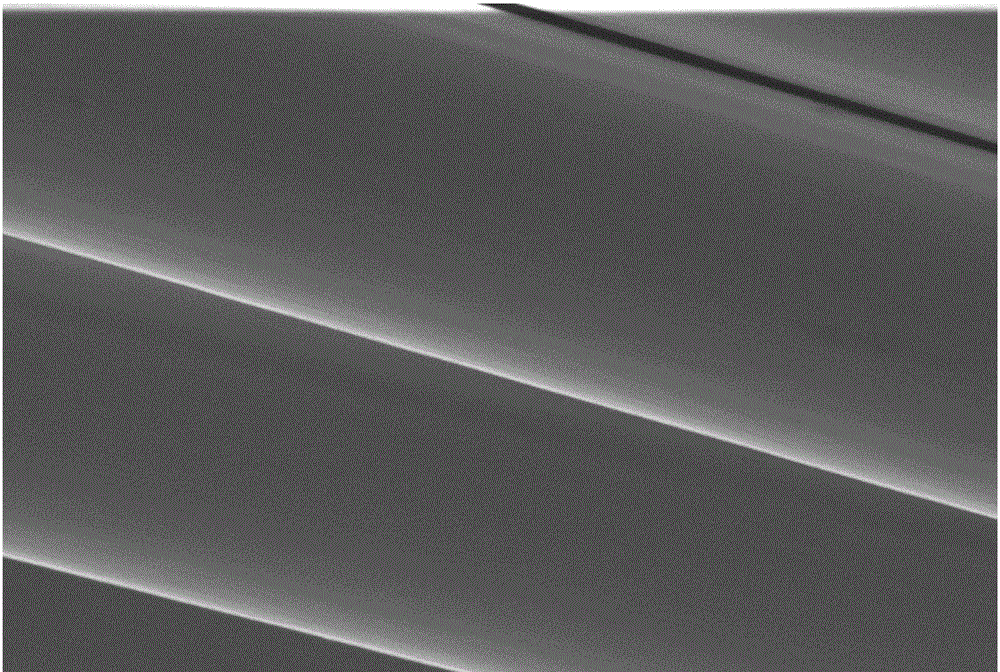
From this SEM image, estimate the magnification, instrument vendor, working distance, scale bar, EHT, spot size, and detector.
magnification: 10 K X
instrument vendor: FEI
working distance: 2.1 mm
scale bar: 10000 nm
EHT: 5 kV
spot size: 2.5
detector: ETD